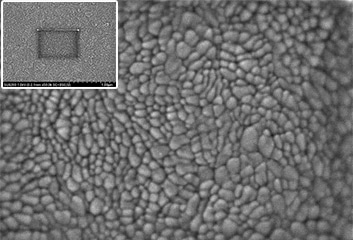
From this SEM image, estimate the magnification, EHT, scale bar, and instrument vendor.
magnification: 150 K X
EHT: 1 kV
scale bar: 300 nm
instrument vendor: Hitachi